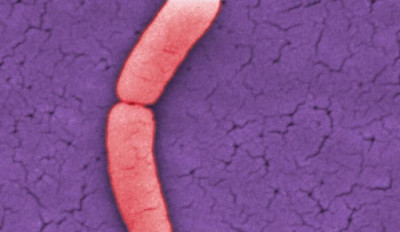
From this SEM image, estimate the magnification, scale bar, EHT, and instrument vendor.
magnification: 25 K X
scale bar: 1000 nm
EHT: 30 kV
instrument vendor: FEI